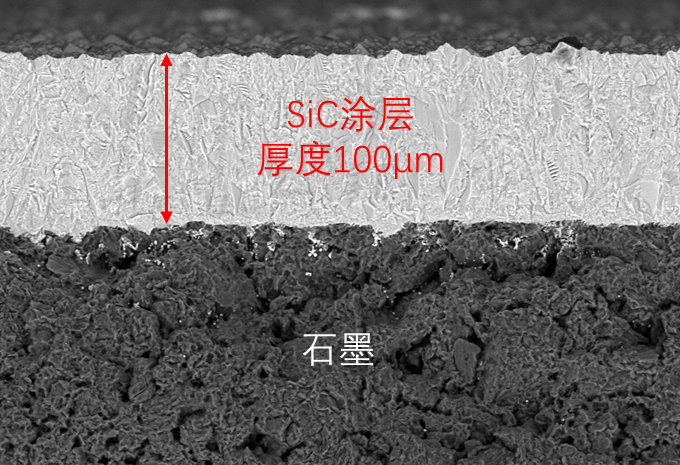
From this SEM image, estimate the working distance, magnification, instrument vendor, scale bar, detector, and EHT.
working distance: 9.2 mm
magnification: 0.3 K X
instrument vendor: Zeiss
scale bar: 20000 nm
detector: BSD1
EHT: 15 kV